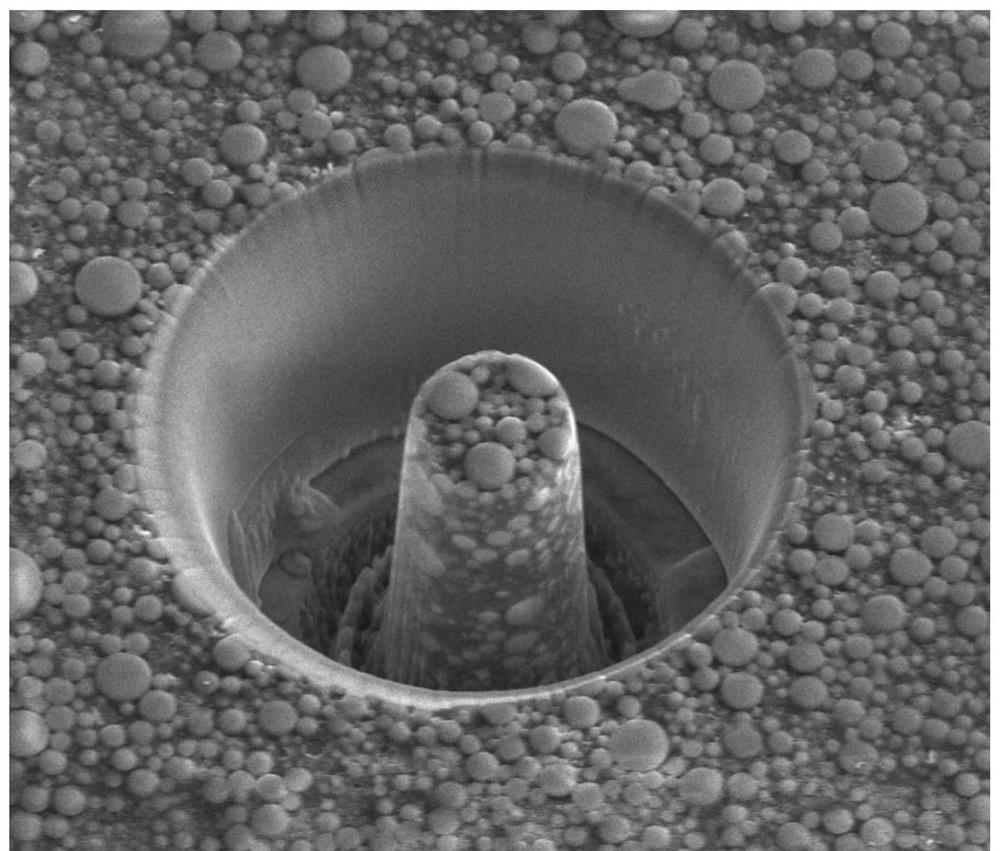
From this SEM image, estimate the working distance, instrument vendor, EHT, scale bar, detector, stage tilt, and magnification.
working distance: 4 mm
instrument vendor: FEI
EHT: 10 kV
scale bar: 20000 nm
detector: ETD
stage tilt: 30°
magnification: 1.999 K X